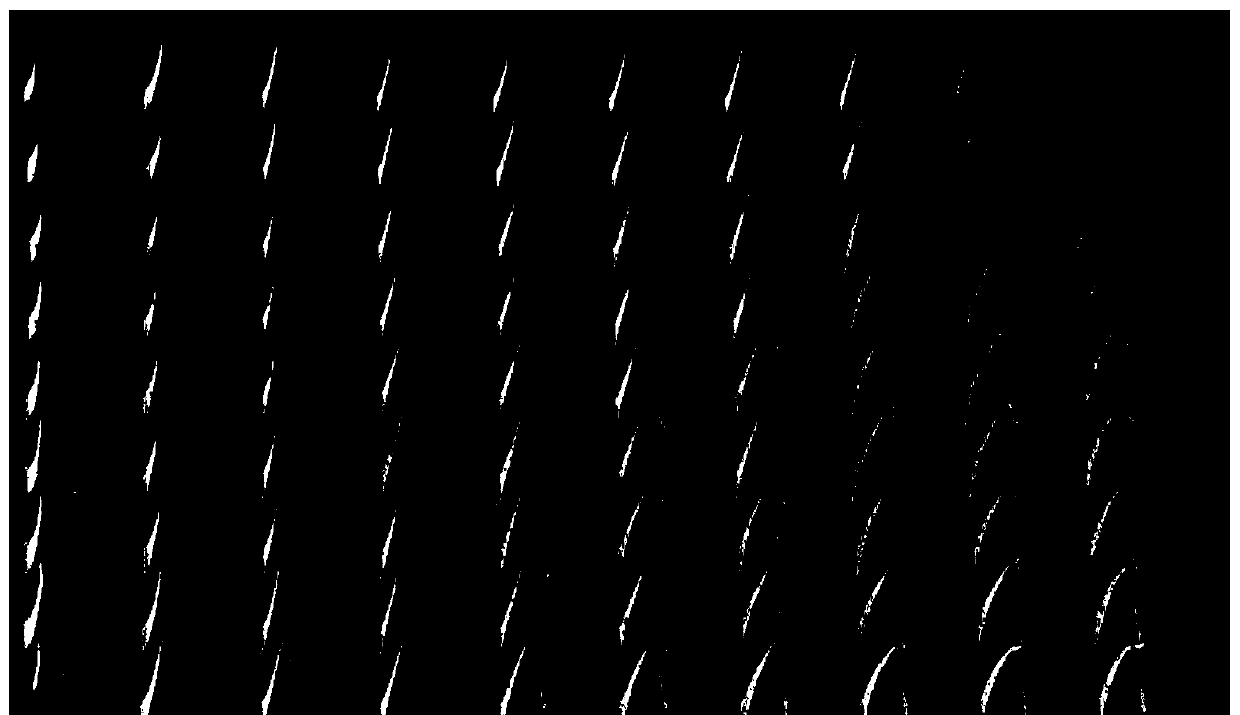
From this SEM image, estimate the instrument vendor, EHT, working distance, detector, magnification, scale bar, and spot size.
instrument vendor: FEI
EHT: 15 kV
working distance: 17 mm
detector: SE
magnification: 0.064 K X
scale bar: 1000000 nm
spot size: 4